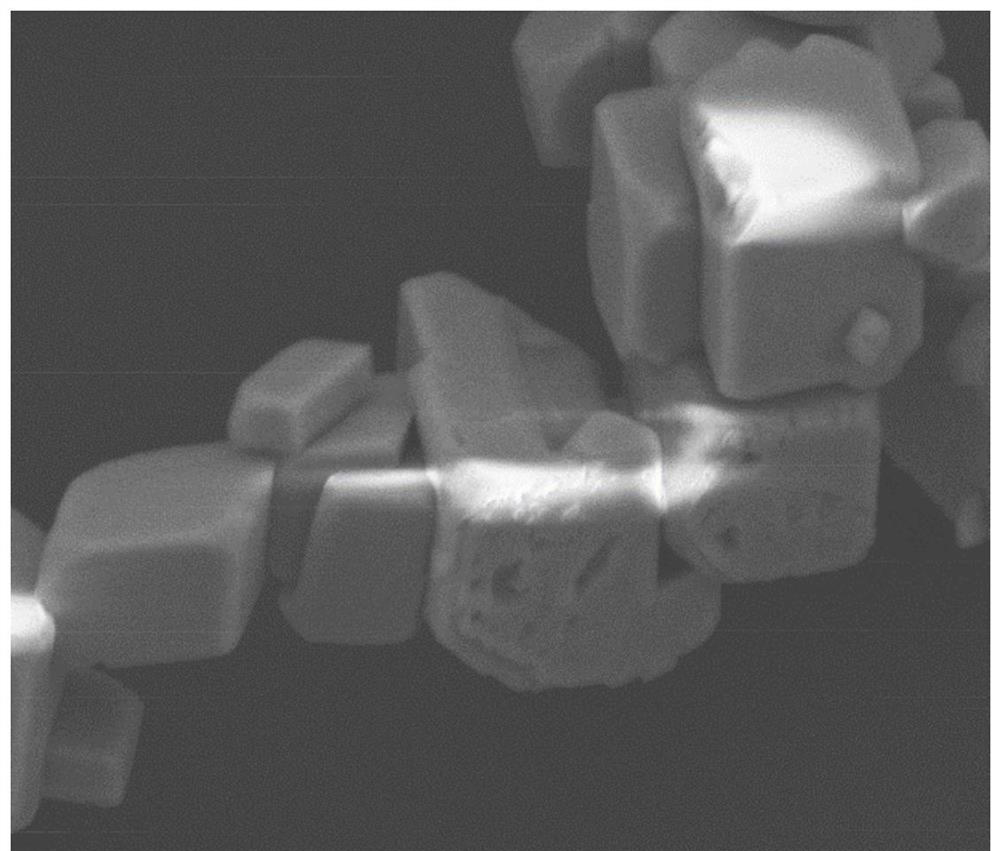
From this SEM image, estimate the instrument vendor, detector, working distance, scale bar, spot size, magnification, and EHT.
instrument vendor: FEI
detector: ETD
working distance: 8.1 mm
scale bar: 10000 nm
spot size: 4.5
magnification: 8 K X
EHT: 12.5 kV